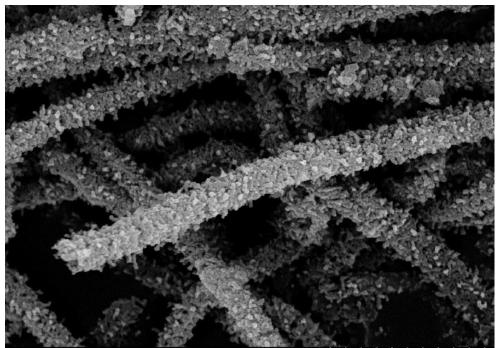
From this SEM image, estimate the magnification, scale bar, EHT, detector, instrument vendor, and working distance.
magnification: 20 K X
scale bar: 2000 nm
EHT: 15 kV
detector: SE(U)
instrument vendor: Hitachi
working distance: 8.4 mm